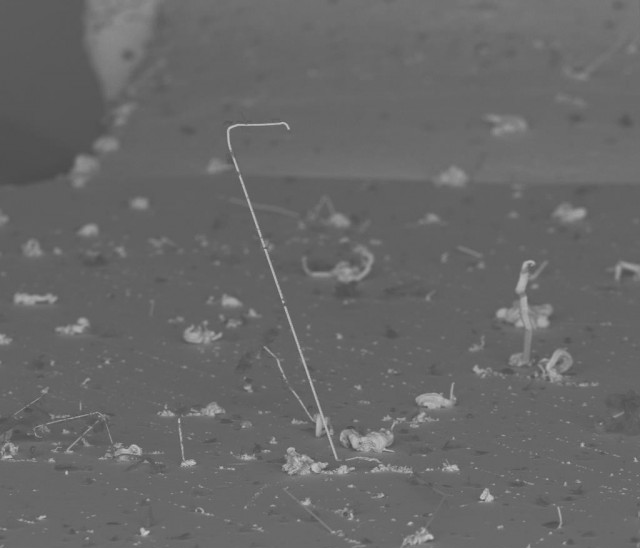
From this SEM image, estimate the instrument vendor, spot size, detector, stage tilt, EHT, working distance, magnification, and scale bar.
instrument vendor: FEI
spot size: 3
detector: BSE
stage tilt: -8.4°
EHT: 20 kV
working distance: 19.5 mm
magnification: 0.199 K X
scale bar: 300000 nm